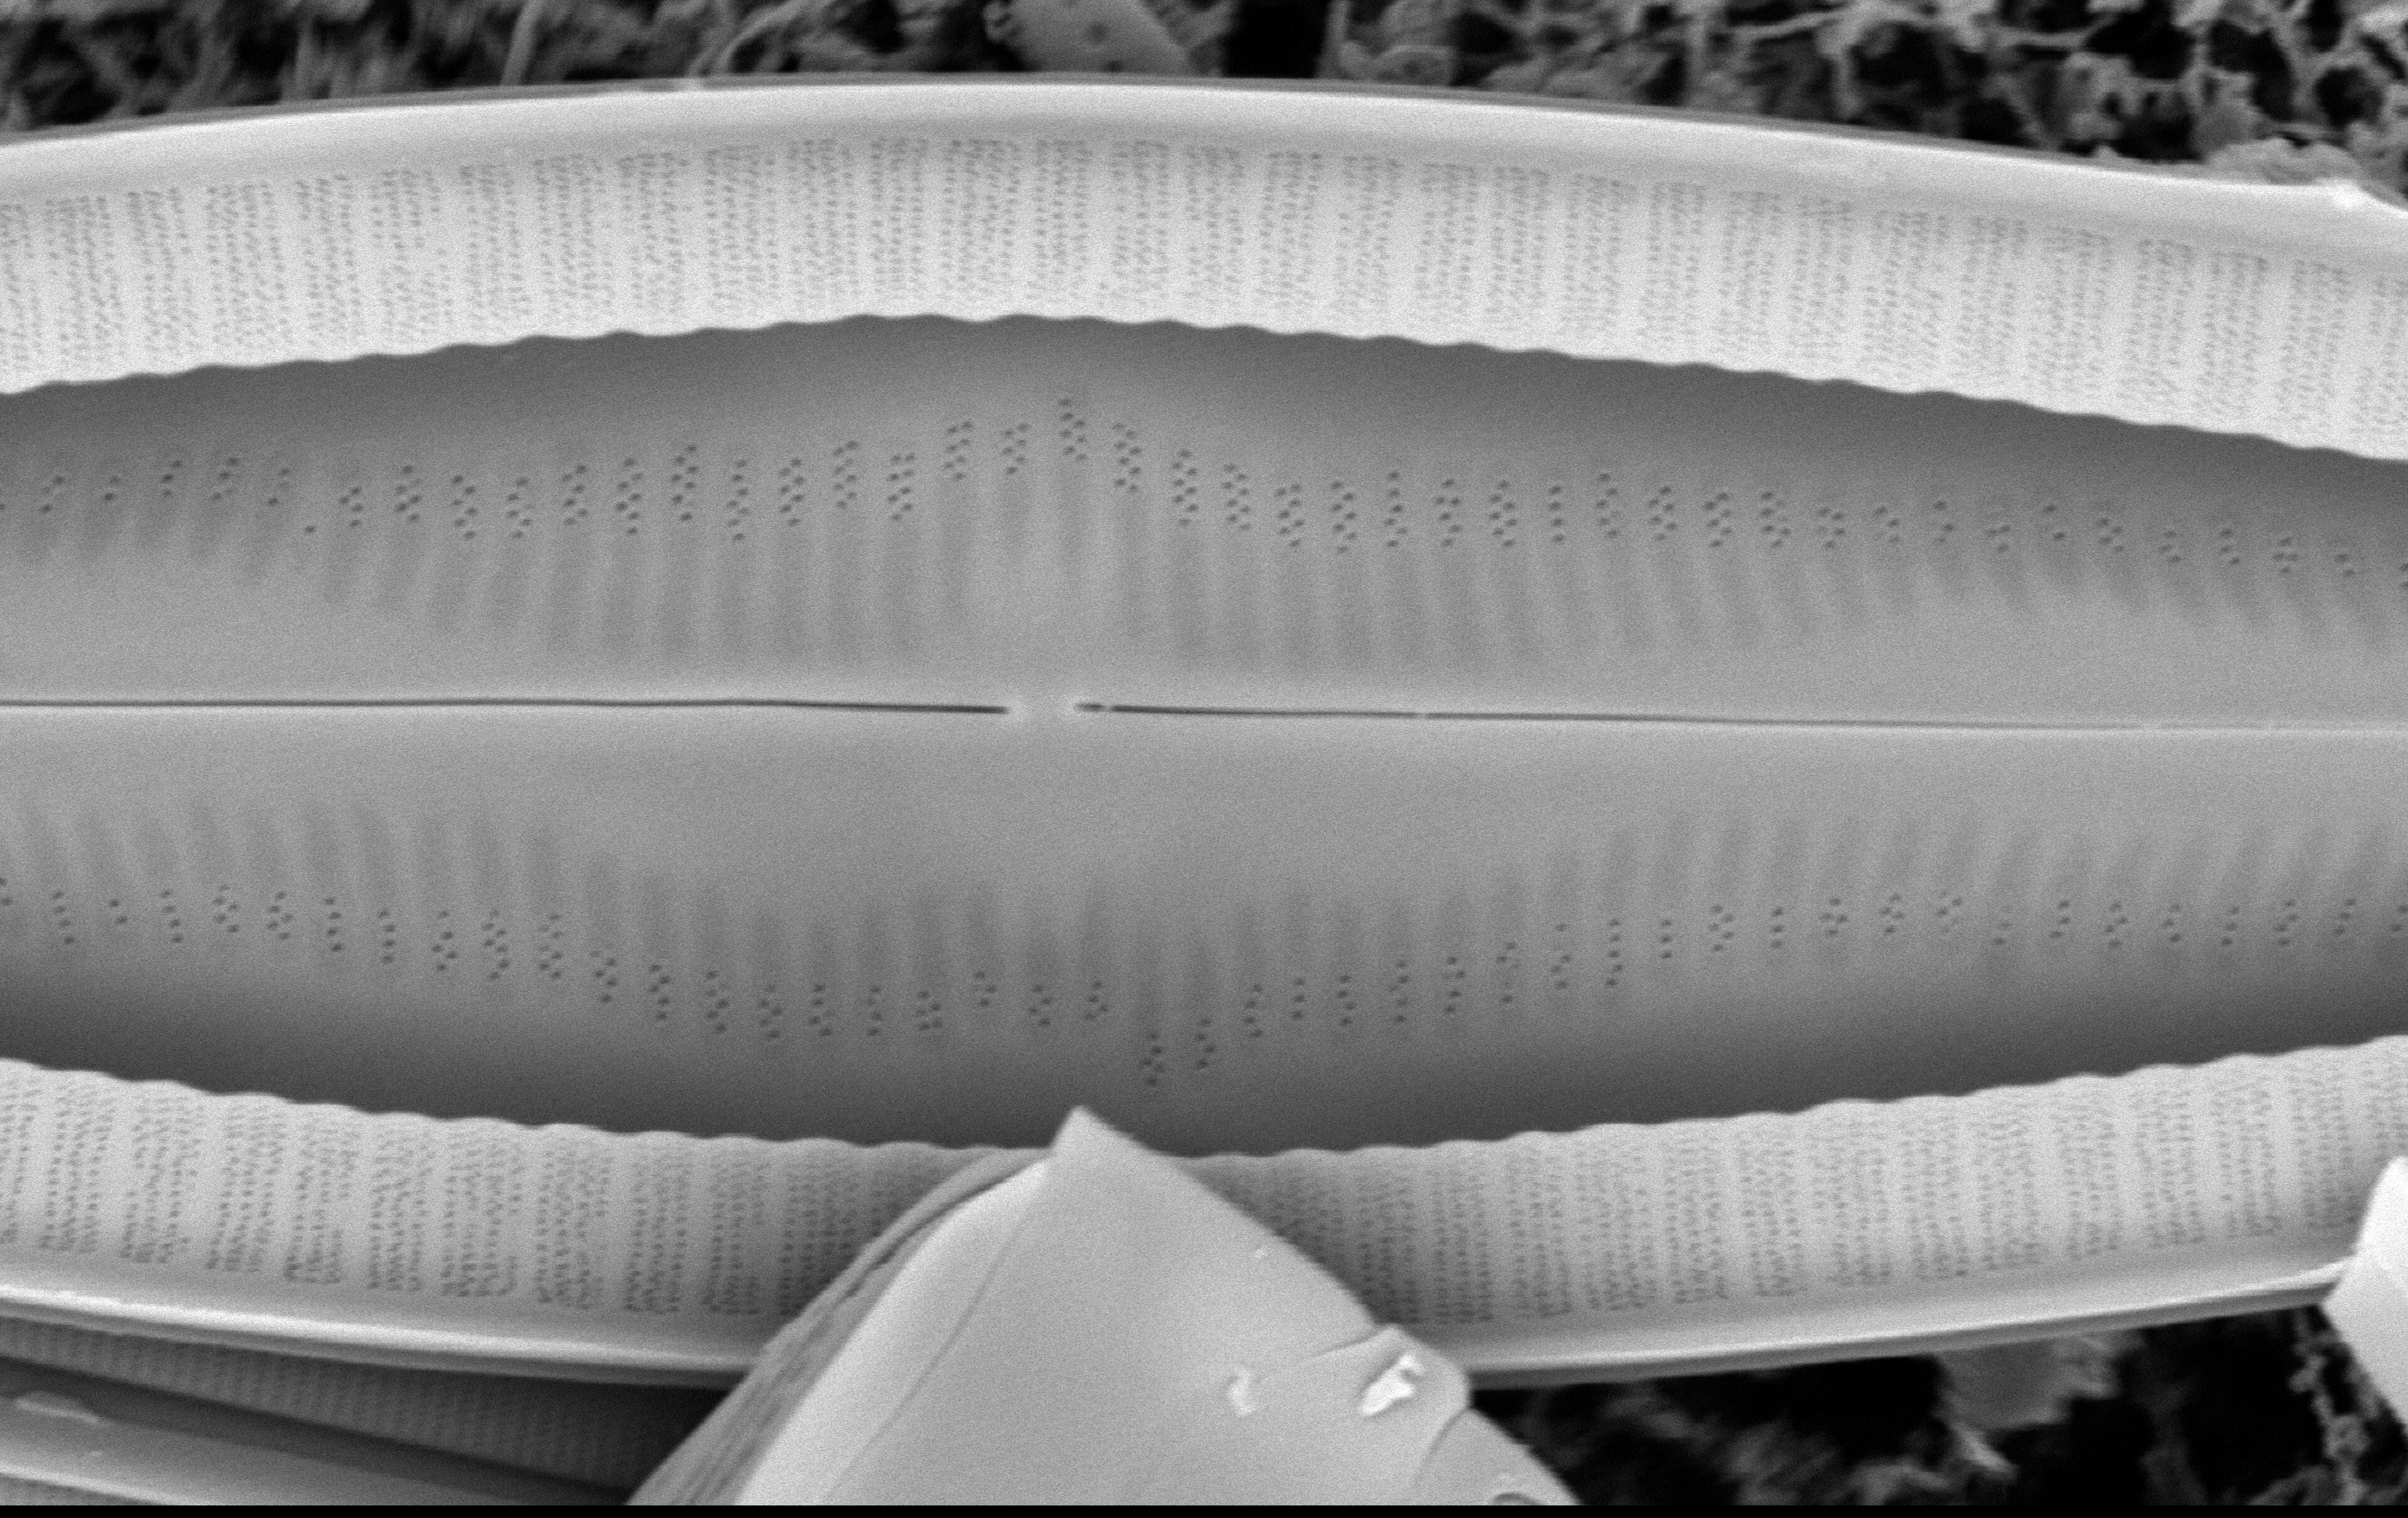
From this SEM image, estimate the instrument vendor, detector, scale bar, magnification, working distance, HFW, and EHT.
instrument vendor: Tescan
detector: BSD Full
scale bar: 8000 nm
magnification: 19 K X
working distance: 10.226 mm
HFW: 27.1 µm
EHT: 10 kV